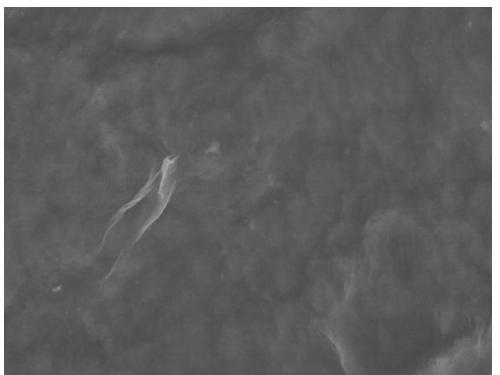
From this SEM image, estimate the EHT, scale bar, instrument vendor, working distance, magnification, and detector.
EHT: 5 kV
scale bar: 2000 nm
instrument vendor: Hitachi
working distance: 9.1 mm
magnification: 20 K X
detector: SE(M,LA0)